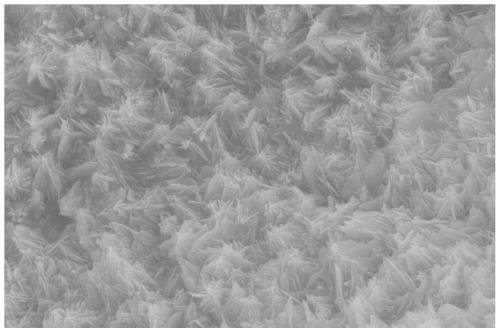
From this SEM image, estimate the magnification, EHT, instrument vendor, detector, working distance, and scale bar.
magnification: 10 K X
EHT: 20 kV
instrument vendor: Zeiss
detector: SE2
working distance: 5.9 mm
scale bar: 1000 nm